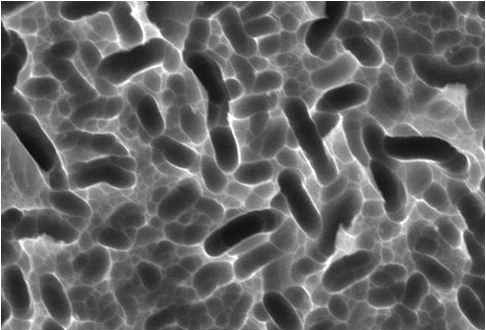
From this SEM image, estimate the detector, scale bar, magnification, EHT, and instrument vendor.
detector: SEI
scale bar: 5000 nm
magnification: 3 K X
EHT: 20 kV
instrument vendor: JEOL